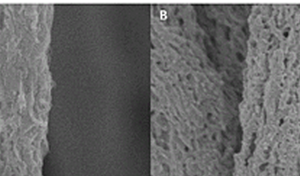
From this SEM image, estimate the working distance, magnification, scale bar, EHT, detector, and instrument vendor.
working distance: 7.1 mm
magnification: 50 K X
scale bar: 1000 nm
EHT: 1 kV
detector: SE(U)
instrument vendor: Hitachi